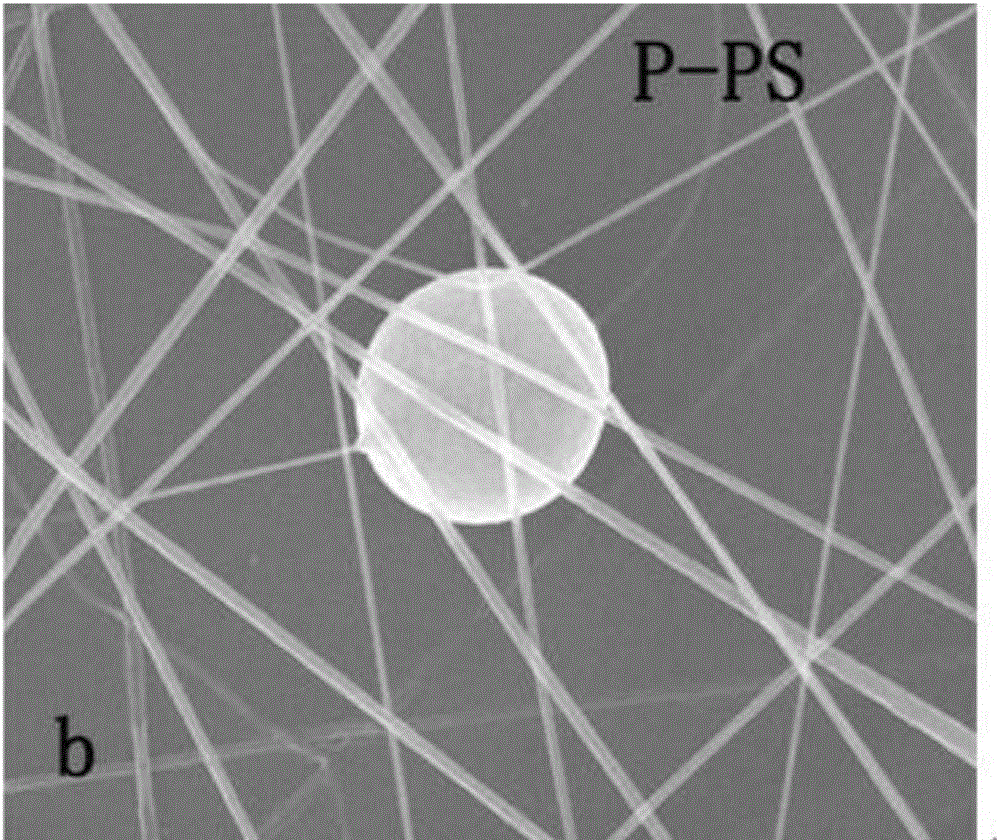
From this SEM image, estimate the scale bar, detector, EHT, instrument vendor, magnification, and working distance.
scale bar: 10000 nm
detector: ETD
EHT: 20 kV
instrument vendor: FEI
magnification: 10 K X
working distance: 10.8 mm